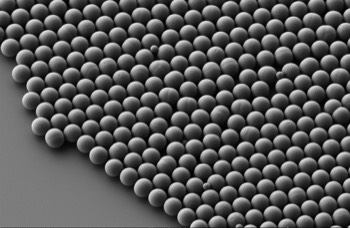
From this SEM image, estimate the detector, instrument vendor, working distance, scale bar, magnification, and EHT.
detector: SE2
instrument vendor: Zeiss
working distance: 3.6 mm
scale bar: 1000 nm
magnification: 5 K X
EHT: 1 kV